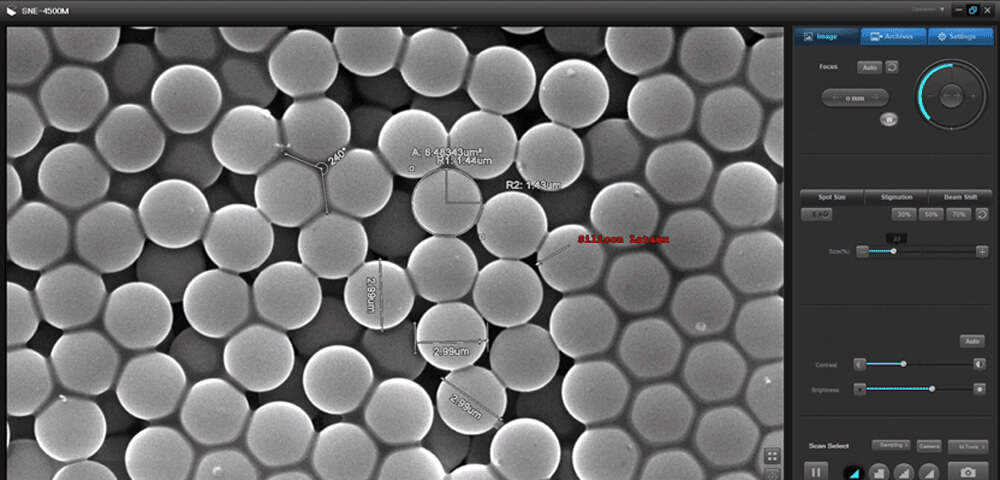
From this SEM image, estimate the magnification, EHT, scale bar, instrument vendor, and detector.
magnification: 10 K X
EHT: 30 kV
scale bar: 10000 nm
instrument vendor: other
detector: SE